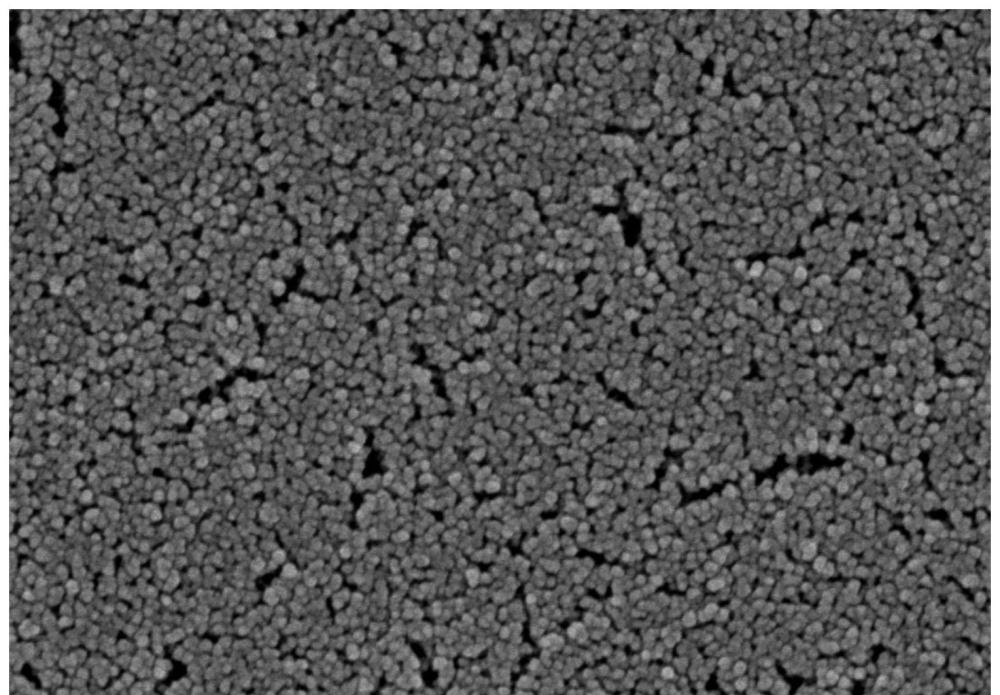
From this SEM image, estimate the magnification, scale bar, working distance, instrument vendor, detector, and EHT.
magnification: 90 K X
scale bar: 500 nm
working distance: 4.4 mm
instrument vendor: Hitachi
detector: SE(UL)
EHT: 3 kV